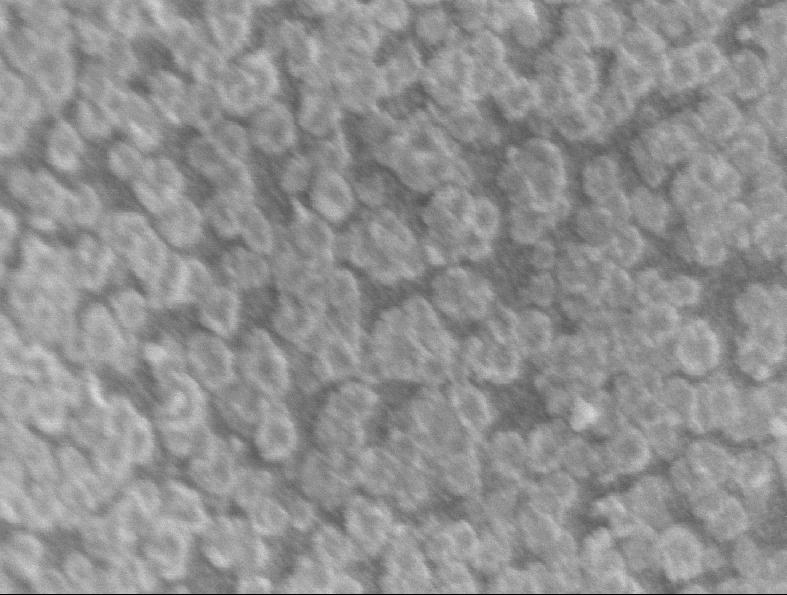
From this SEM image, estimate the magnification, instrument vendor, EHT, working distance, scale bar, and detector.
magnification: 110 K X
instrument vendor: JEOL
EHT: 1 kV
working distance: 2 mm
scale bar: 100 nm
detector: SEI